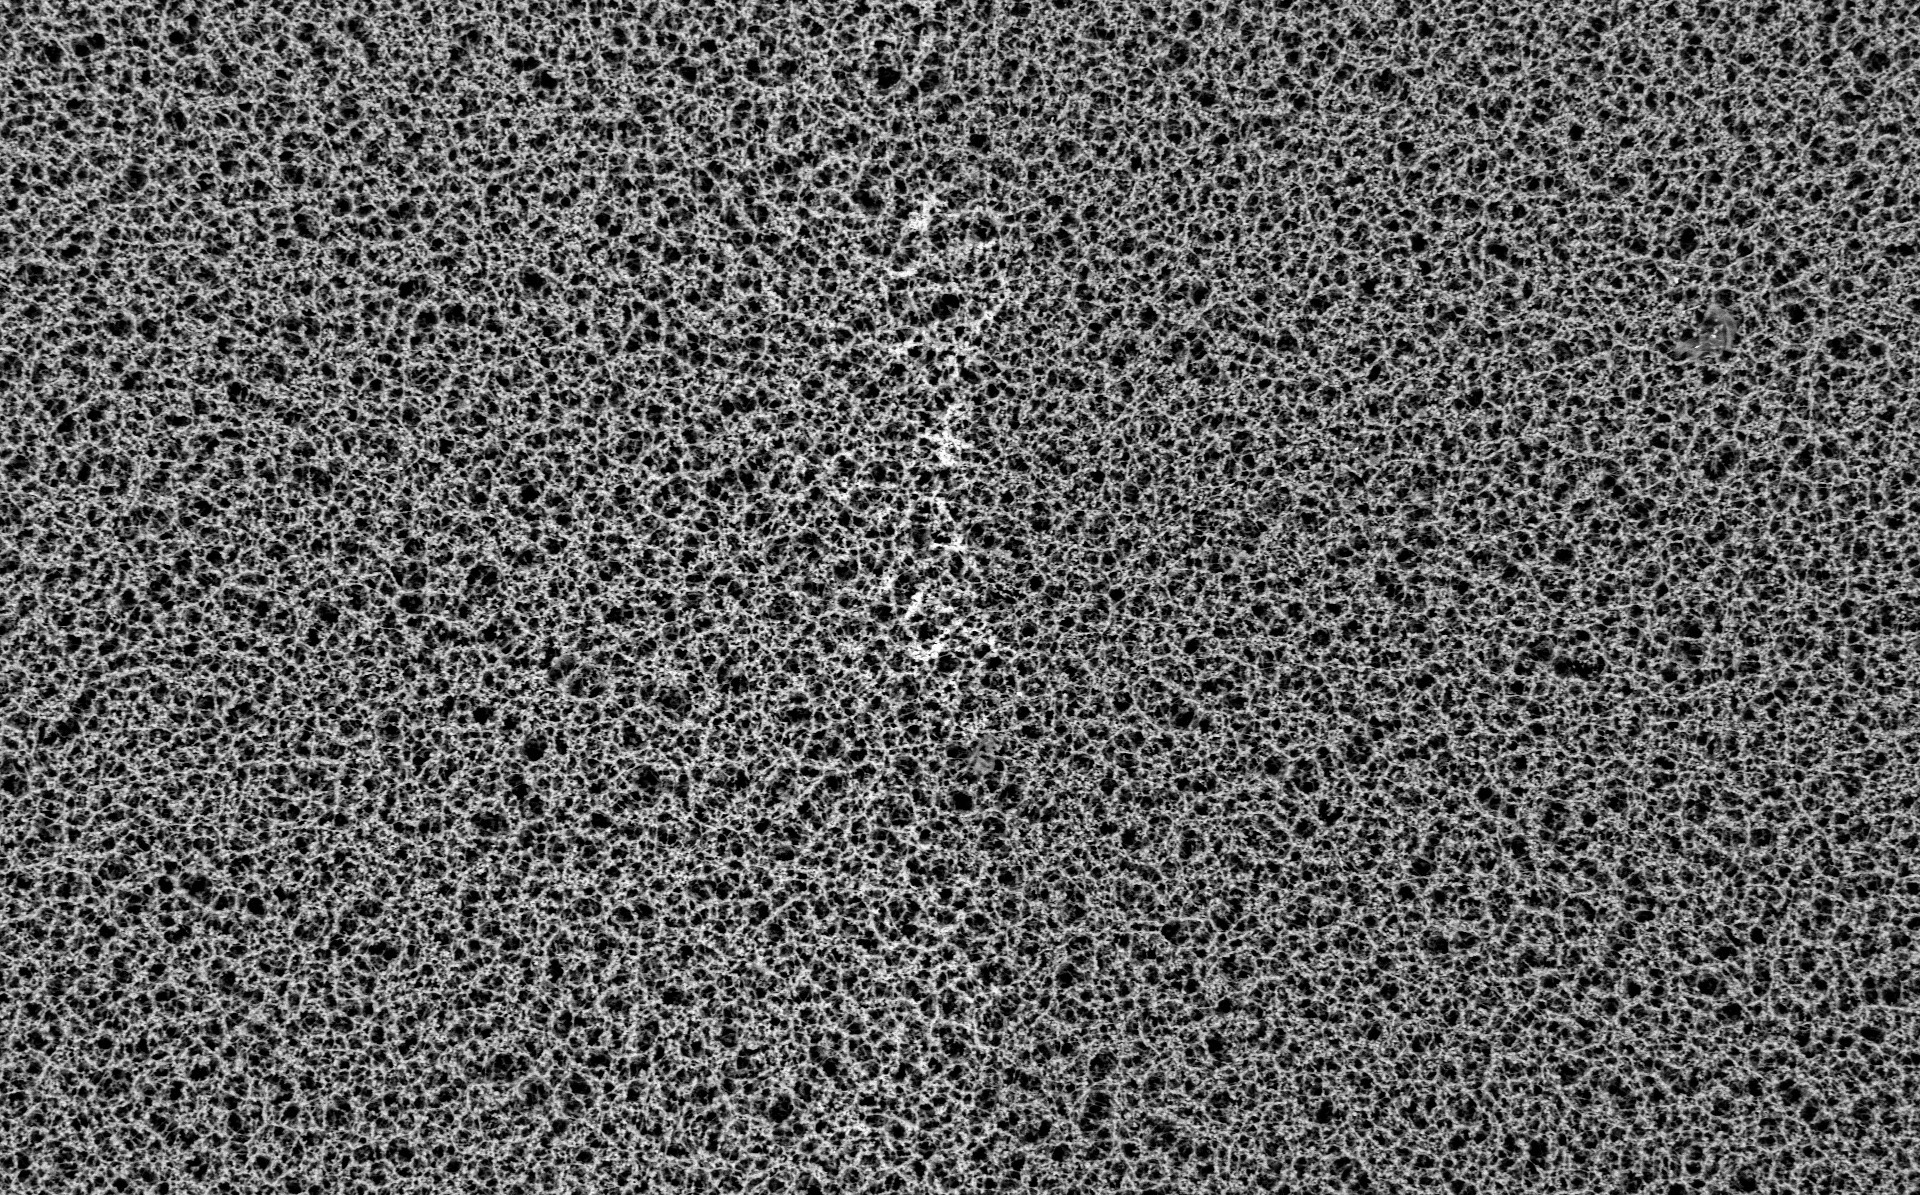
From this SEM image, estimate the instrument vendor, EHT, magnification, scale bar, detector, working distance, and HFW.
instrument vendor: Thermo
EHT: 15 kV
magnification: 0.13 K X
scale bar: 300000 nm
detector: BSD Full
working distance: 8.194 mm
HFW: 979 µm